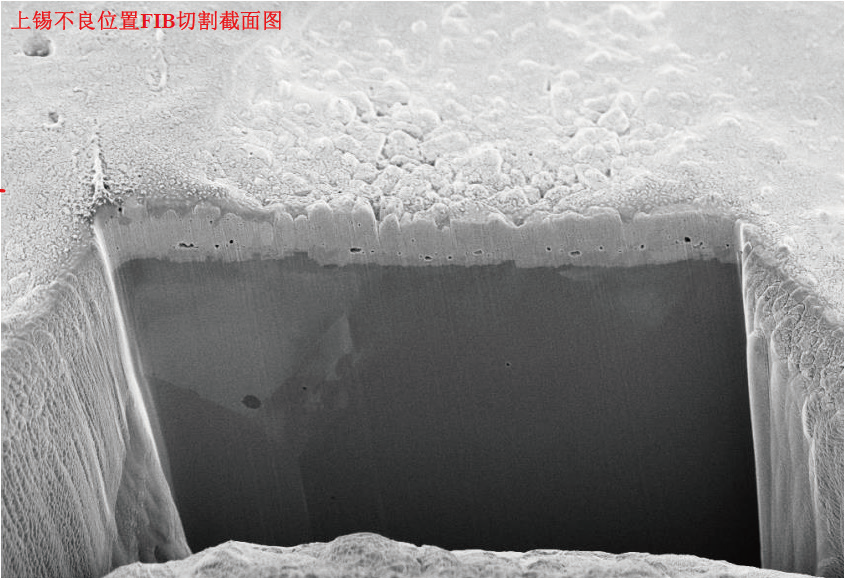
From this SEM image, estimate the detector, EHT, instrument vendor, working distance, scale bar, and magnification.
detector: InLens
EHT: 3 kV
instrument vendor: Zeiss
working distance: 2.9 mm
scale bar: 2000 nm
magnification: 5.71 K X